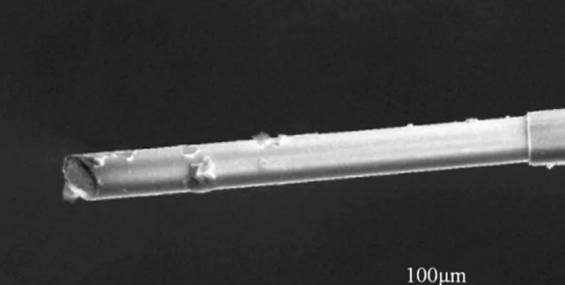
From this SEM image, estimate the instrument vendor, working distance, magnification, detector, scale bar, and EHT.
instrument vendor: FEI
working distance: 14.2 mm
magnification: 0.5 K X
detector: SE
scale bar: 100000 nm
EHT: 20 kV